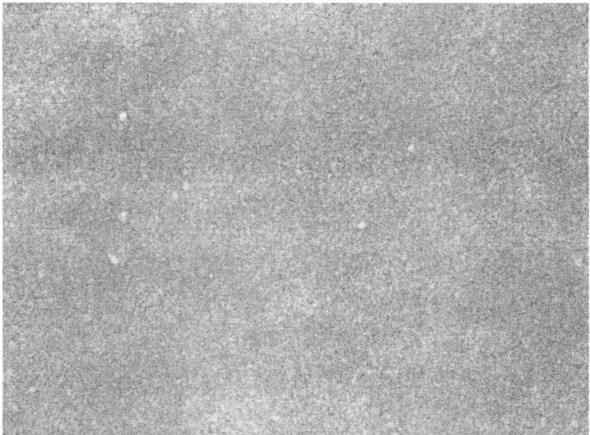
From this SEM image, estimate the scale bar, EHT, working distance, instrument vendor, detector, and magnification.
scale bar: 1000 nm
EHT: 30 kV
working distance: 7.5 mm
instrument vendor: JEOL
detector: SEI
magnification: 10 K X